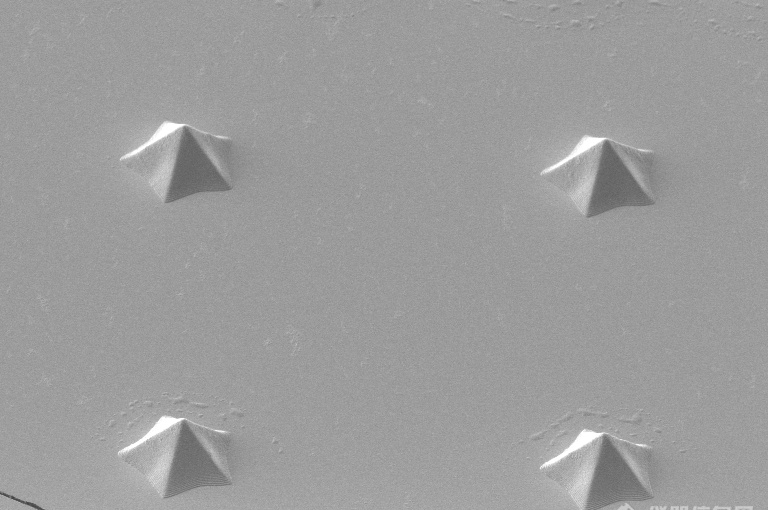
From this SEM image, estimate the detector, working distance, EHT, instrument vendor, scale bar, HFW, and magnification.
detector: ICE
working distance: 3.8 mm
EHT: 2 kV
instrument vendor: FEI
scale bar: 20000 nm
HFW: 82.9 µm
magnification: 2.5 K X